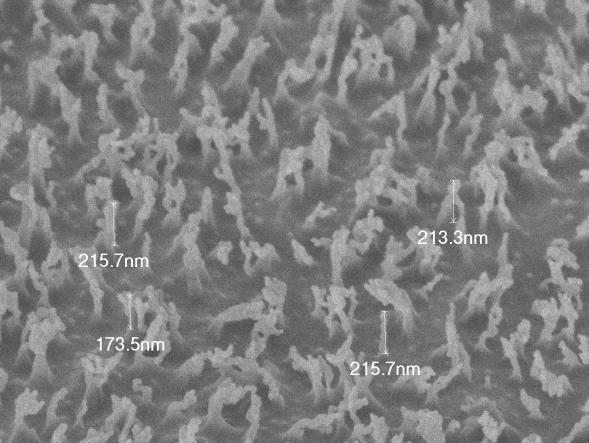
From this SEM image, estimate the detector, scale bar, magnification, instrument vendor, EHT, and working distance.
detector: SEI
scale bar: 100 nm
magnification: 40 K X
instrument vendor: JEOL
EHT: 2 kV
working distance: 4.4 mm